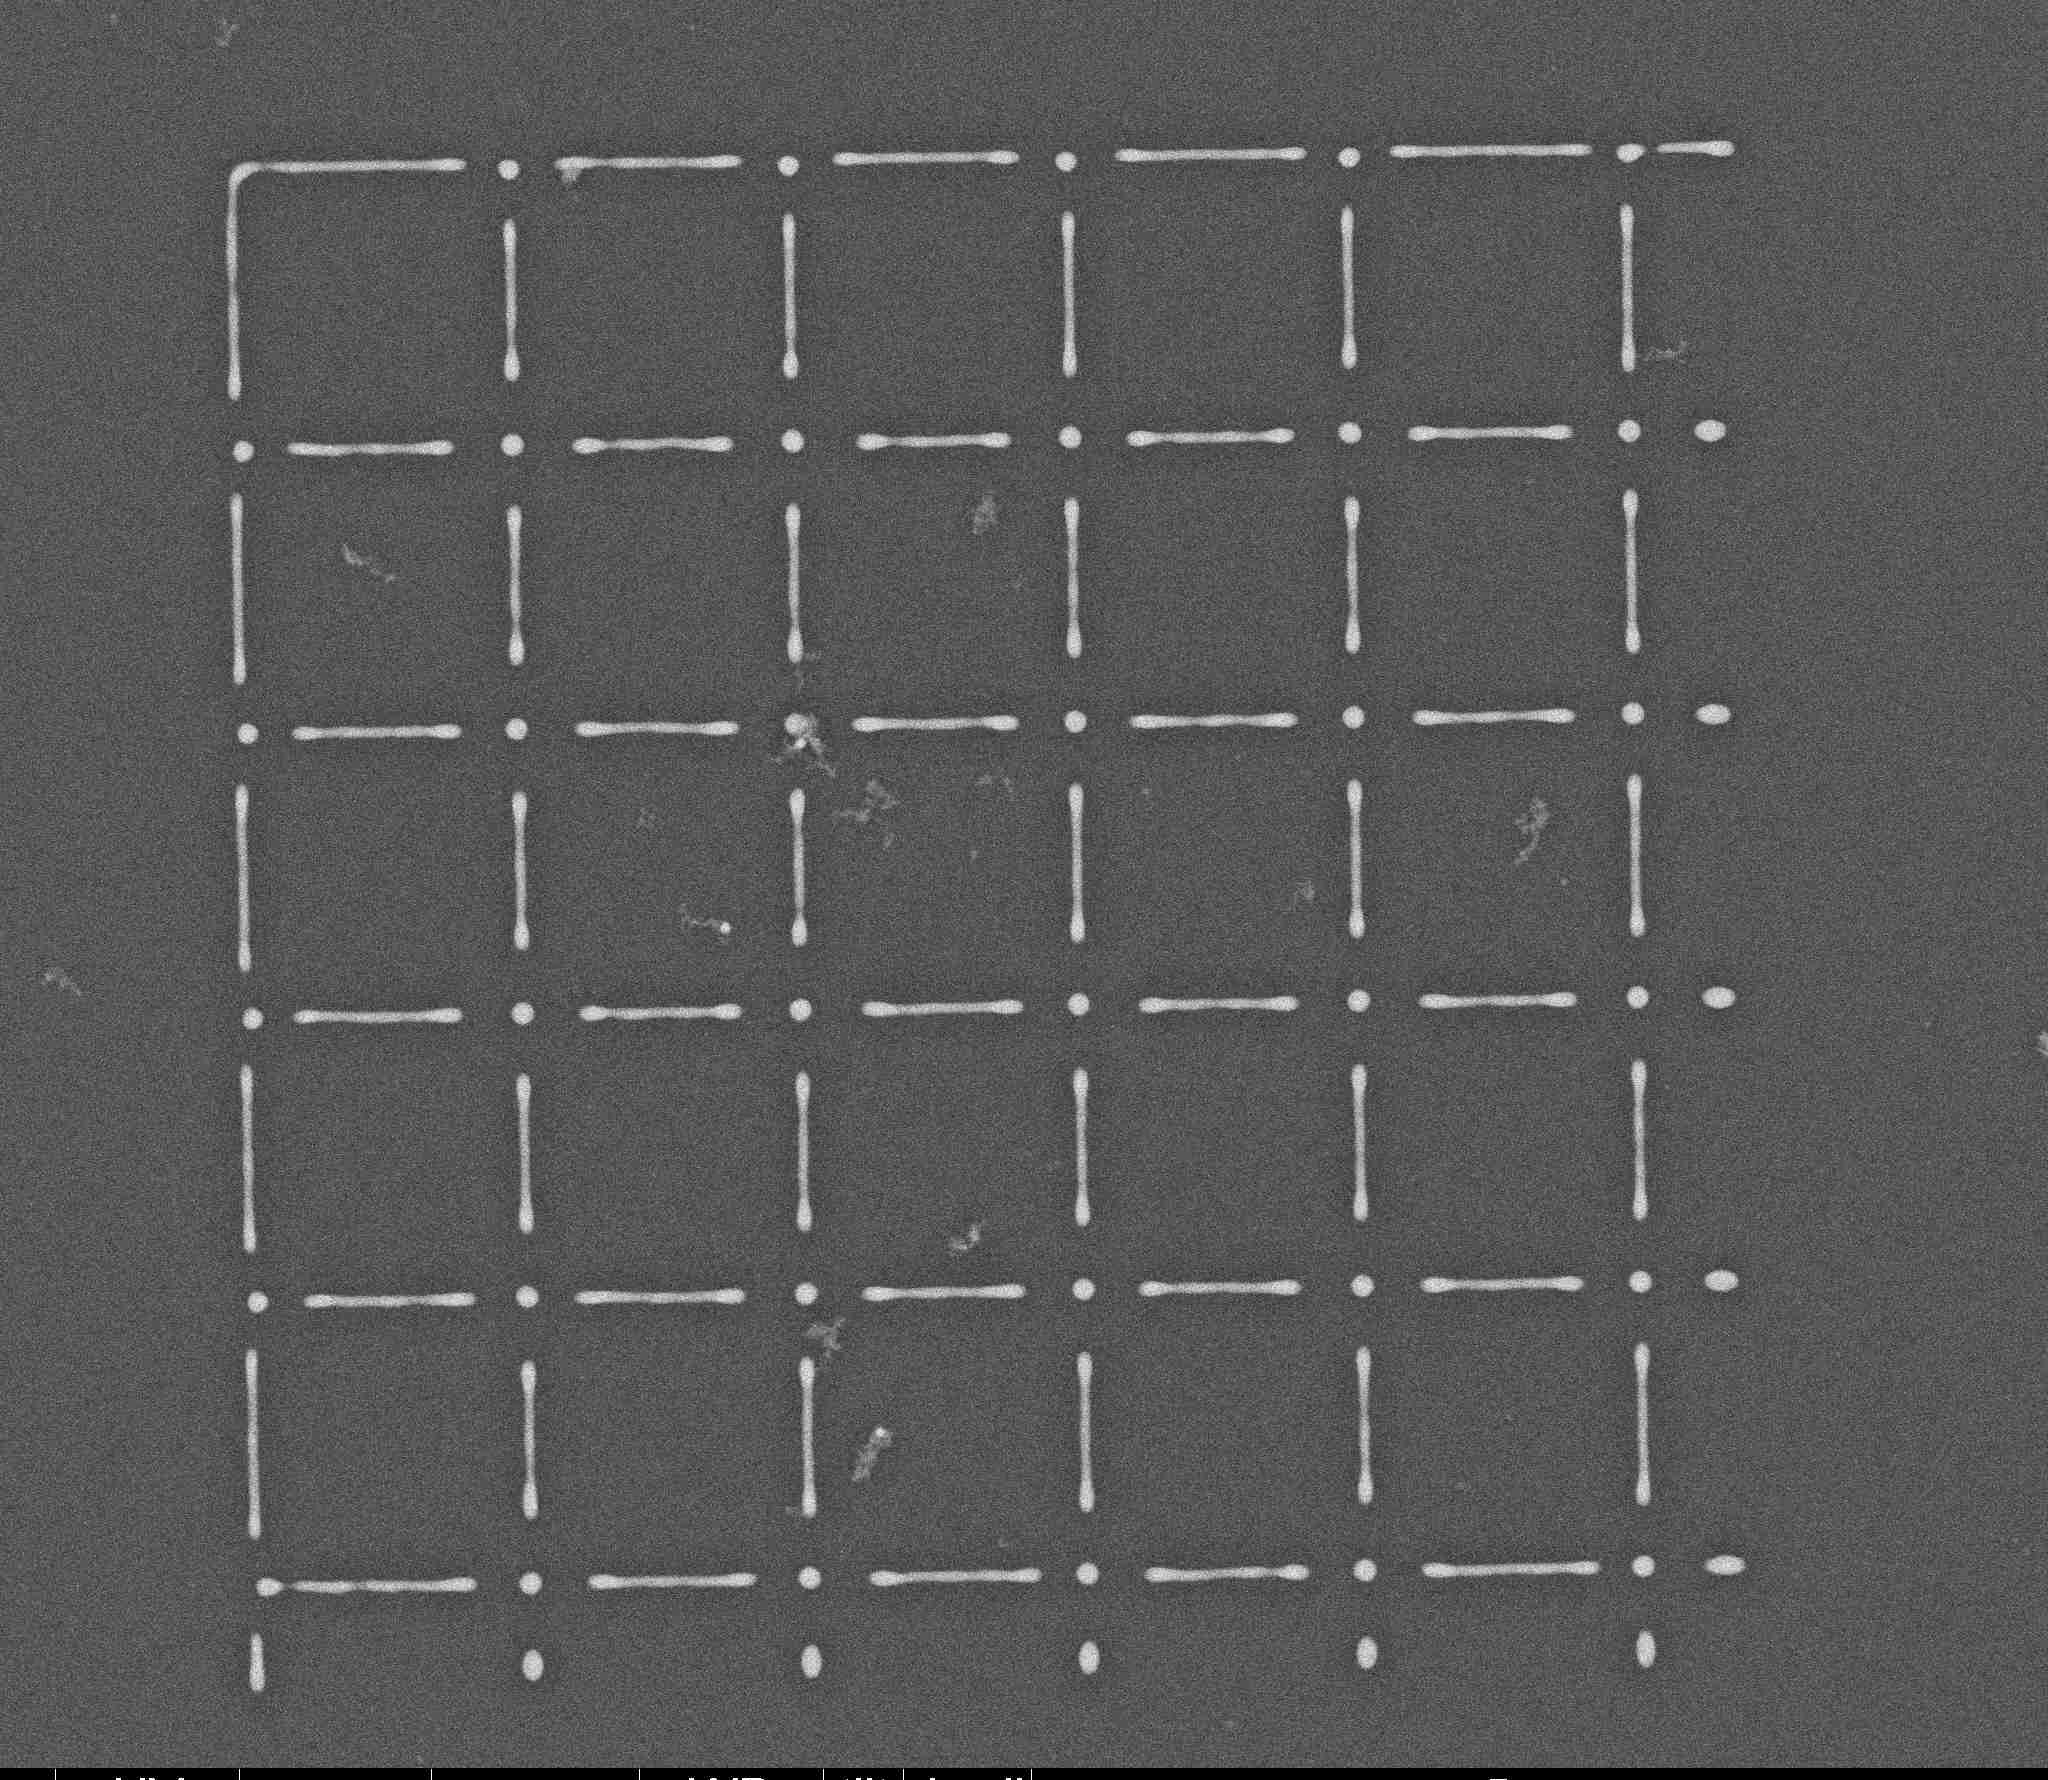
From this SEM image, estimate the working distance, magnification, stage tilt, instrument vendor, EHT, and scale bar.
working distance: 5.3 mm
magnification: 10 K X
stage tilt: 0°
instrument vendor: FEI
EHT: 5 kV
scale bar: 5000 nm